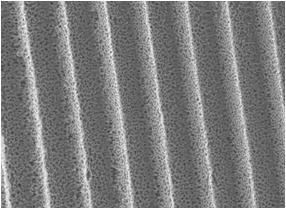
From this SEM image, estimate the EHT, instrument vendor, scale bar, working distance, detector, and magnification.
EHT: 10 kV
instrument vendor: JEOL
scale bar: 10000 nm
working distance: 8 mm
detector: LEI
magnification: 0.8 K X